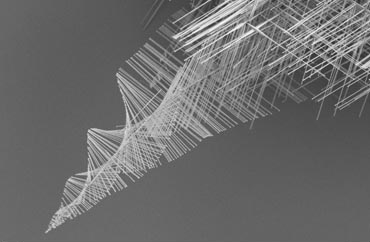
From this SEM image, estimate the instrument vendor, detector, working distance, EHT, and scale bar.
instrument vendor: Zeiss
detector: SE2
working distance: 7 mm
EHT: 1.5 kV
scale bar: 10000 nm